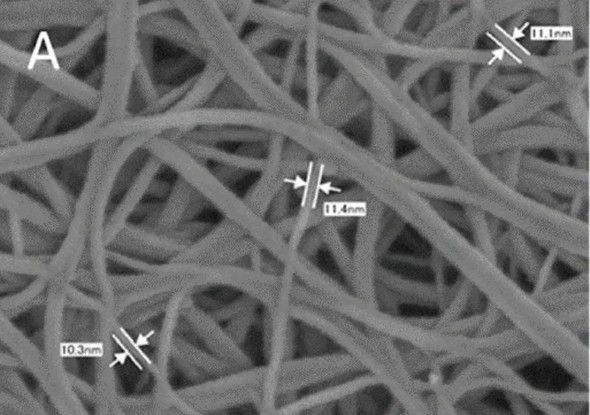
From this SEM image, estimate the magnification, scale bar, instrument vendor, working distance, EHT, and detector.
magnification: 200 K X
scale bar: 100 nm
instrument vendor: JEOL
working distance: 2 mm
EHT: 2 kV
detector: SEI